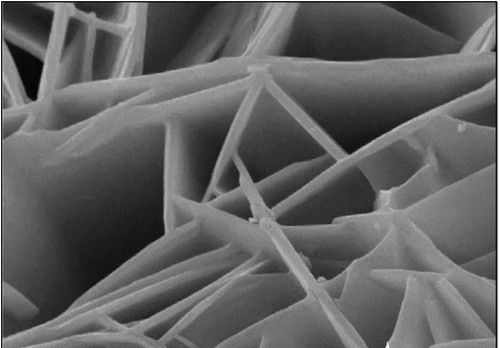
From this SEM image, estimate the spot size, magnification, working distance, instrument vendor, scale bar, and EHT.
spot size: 8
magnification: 30 K X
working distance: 5 mm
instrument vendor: JEOL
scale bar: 1000 nm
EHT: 20 kV